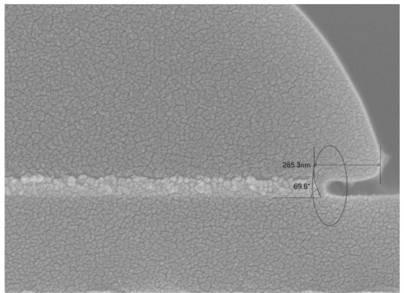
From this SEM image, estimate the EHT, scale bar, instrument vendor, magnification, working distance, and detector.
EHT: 5 kV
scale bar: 100 nm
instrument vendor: JEOL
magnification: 70 K X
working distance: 3 mm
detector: SEI